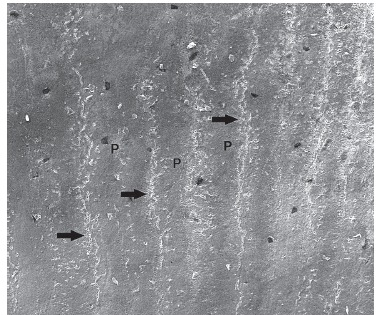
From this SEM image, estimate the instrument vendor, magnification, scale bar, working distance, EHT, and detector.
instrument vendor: FEI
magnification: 0.2 K X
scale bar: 500000 nm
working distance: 10.7 mm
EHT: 30 kV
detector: ETD